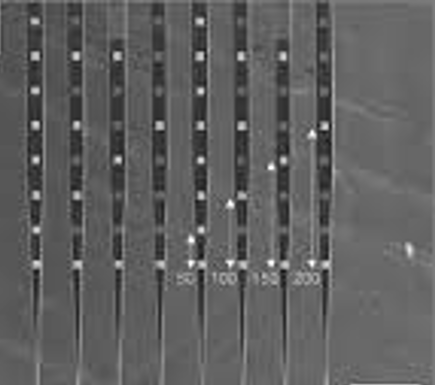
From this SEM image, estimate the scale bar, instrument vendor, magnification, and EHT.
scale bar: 100000 nm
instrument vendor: JEOL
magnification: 0.13 K X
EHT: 15 kV